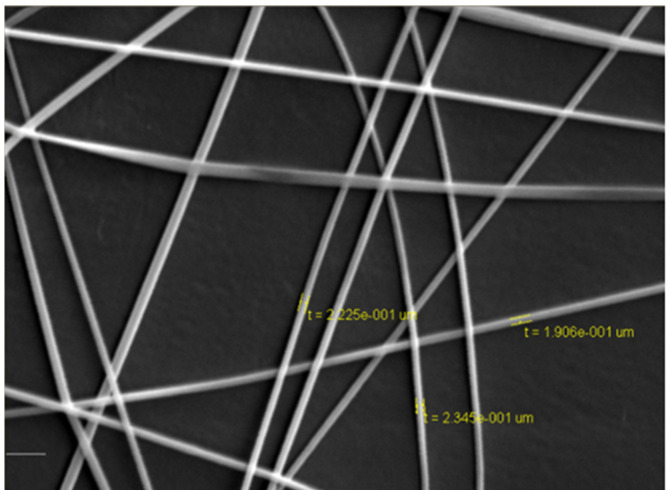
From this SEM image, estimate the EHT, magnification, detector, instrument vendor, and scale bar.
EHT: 10 kV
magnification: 10 K X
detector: SE Detector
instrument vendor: Tescan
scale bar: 10000 nm